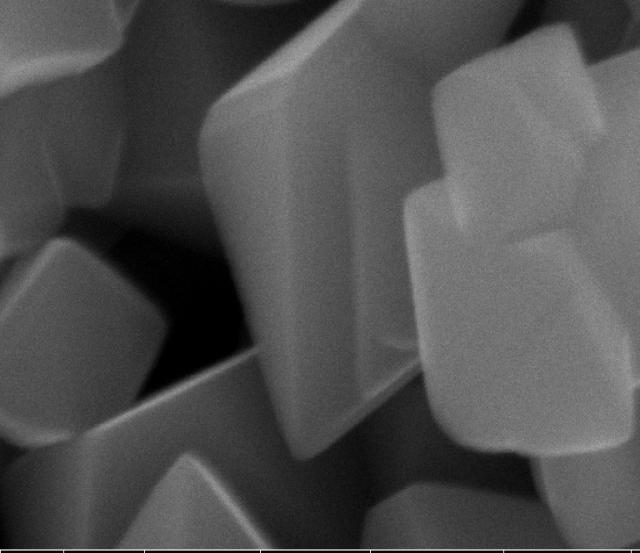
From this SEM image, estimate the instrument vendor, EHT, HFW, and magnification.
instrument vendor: FEI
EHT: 15 kV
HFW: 4.14 µm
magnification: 100 K X